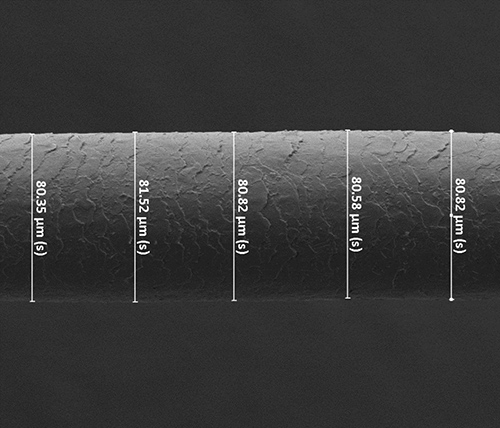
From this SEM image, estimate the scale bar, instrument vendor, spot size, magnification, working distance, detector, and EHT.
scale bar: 100000 nm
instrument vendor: FEI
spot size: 2.5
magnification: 0.528 K X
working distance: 5.8 mm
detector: ETD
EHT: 10 kV